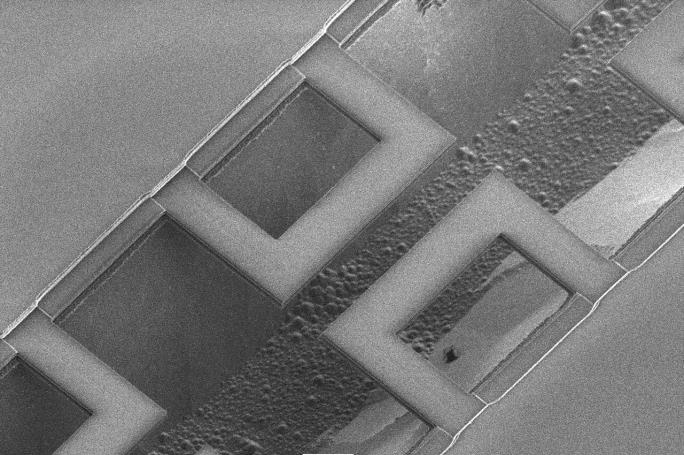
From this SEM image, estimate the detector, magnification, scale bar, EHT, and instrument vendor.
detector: SEI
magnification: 1 K X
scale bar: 10000 nm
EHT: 3 kV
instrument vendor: JEOL